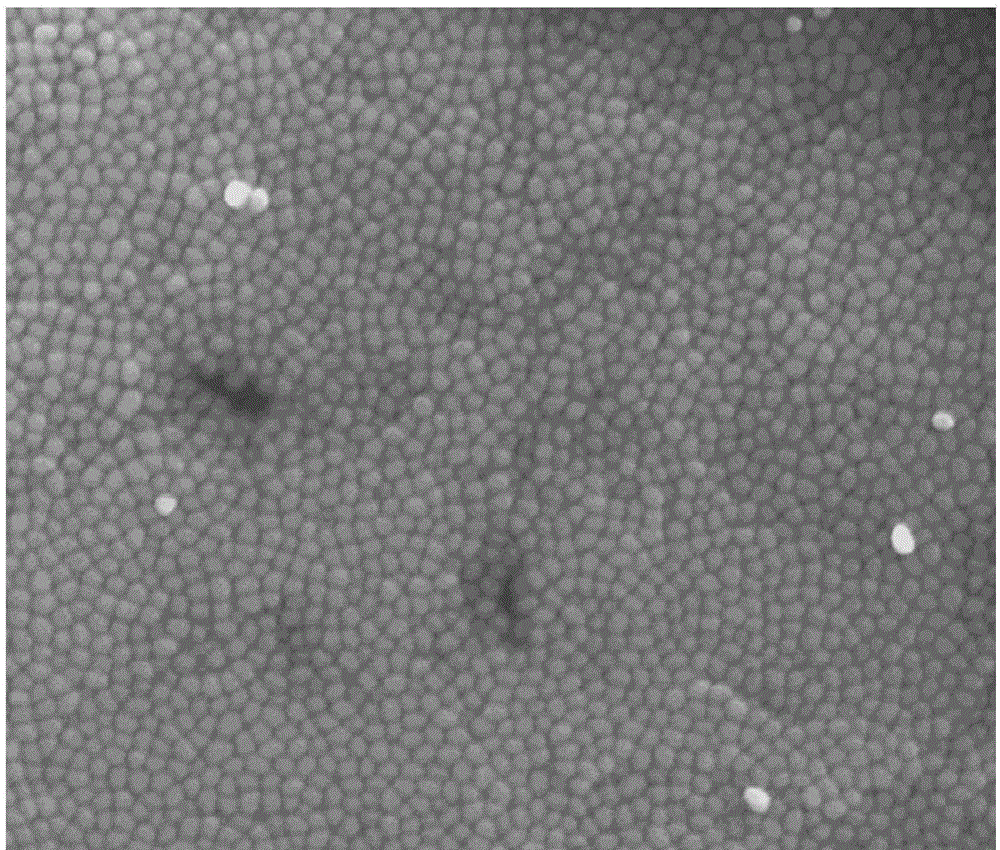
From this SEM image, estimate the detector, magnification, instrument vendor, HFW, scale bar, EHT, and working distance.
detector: TLD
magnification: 100 K X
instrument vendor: FEI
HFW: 2.4 µm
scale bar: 500 nm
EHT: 10 kV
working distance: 5.2 mm